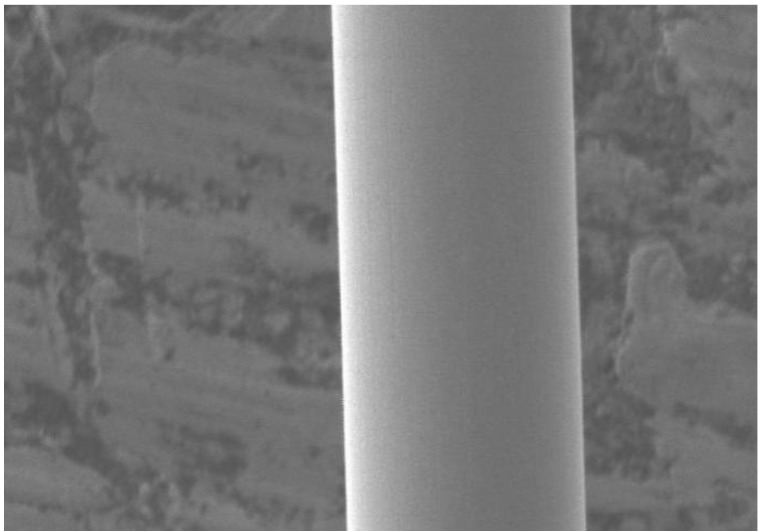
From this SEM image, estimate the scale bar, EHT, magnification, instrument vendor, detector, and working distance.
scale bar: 10000 nm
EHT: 3 kV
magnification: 2 K X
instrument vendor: JEOL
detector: LEI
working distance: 8 mm